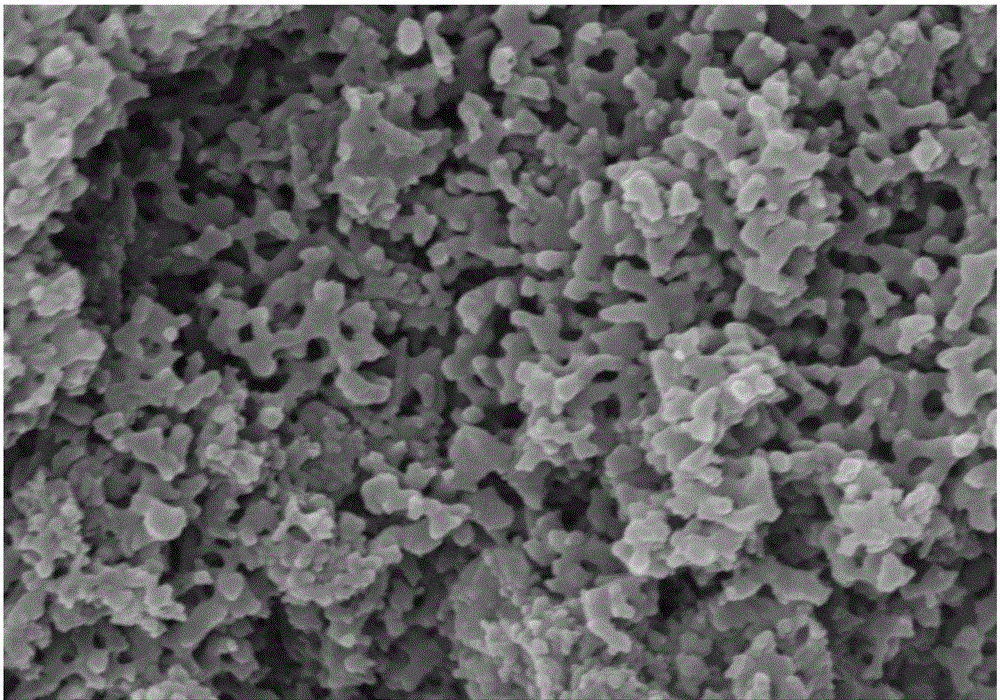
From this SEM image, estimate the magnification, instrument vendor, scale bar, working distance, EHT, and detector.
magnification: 50 K X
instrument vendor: Hitachi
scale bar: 1000 nm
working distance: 7.7 mm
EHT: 15 kV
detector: SE(M,LA100)